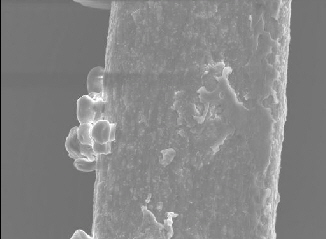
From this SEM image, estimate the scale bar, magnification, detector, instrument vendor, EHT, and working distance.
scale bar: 10000 nm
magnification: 2 K X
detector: SEI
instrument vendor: JEOL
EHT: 10 kV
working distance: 8.9 mm